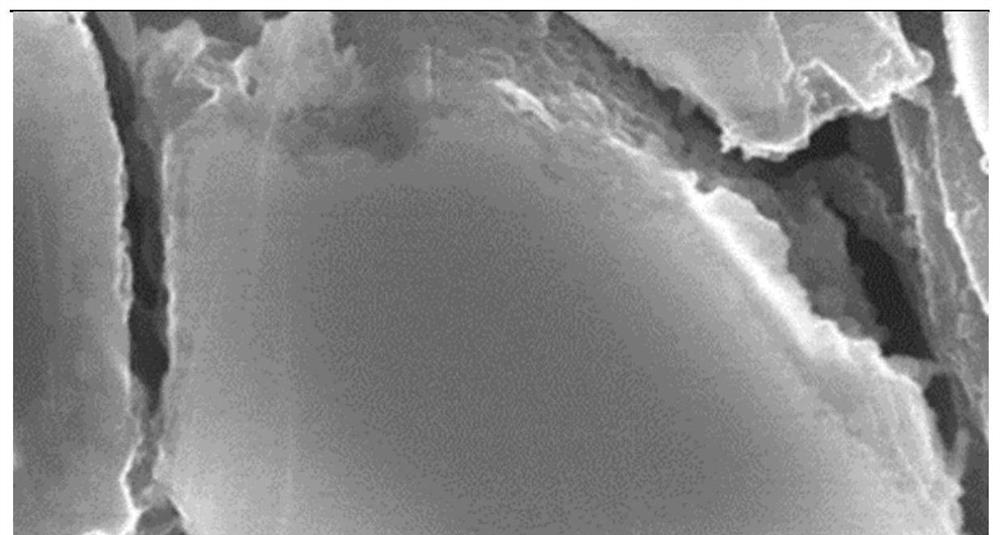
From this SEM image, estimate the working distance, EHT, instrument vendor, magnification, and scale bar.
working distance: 15 mm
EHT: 15 kV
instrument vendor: other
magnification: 10 K X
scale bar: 2000 nm